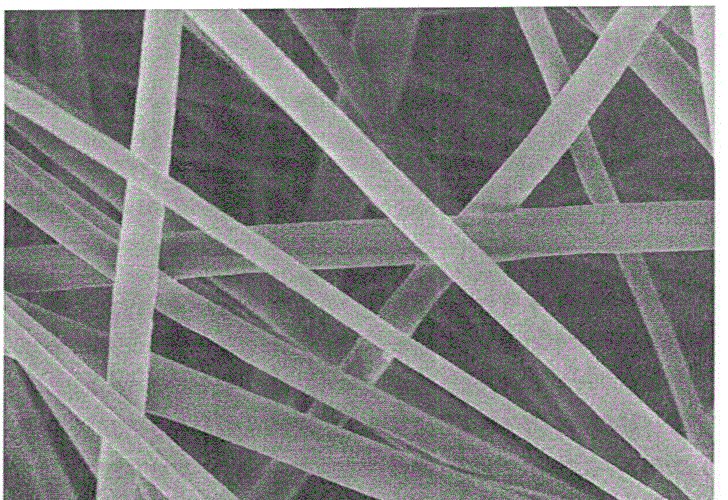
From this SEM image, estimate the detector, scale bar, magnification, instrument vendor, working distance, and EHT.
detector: SE(M)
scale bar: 5000 nm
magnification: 10 K X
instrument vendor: Hitachi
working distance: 6.5 mm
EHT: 1 kV